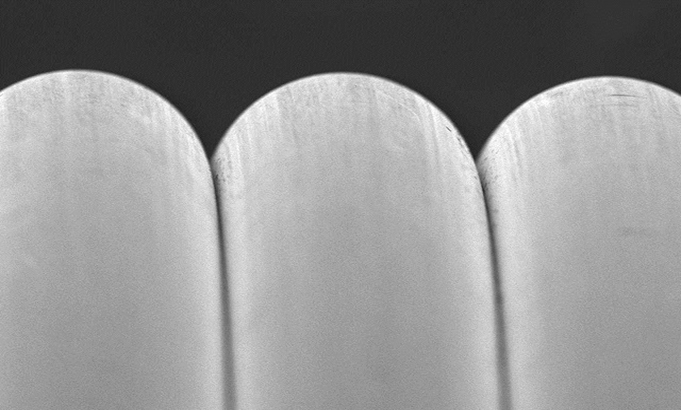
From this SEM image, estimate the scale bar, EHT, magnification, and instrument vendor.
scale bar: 50000 nm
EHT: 10 kV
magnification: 0.5 K X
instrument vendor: JEOL